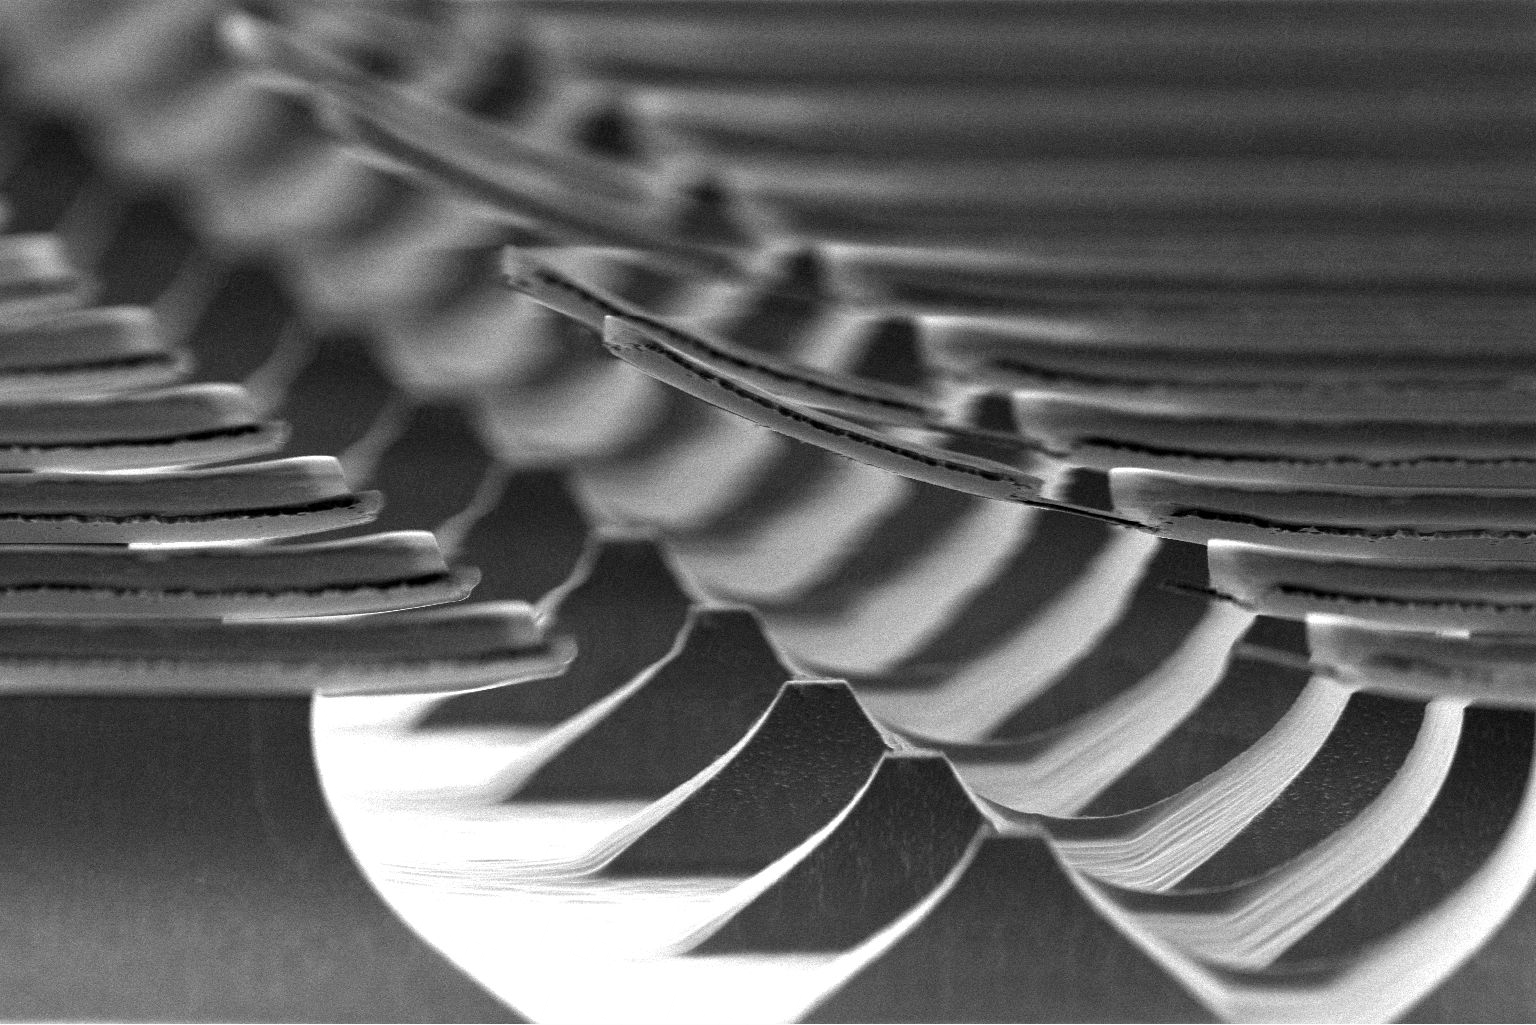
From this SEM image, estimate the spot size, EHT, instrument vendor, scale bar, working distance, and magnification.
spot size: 4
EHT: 5 kV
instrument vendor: FEI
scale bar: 50000 nm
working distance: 3.2 mm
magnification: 1.5 K X